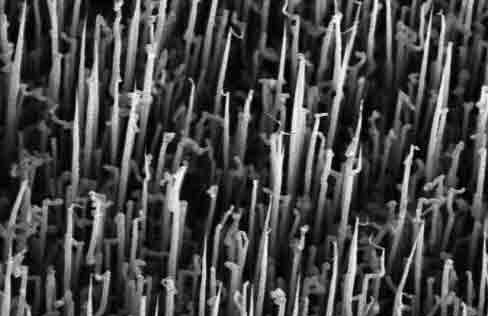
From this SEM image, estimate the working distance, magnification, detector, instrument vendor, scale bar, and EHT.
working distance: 9.1 mm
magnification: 0.8 K X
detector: SE(M)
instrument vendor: Hitachi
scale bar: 50000 nm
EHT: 20 kV